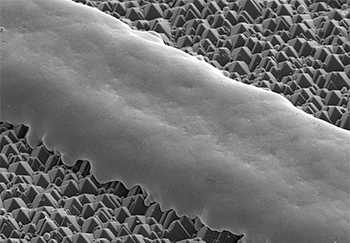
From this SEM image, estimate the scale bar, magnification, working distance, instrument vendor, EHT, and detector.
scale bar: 100000 nm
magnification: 0.5 K X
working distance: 14 mm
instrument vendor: Hitachi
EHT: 10 kV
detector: SE